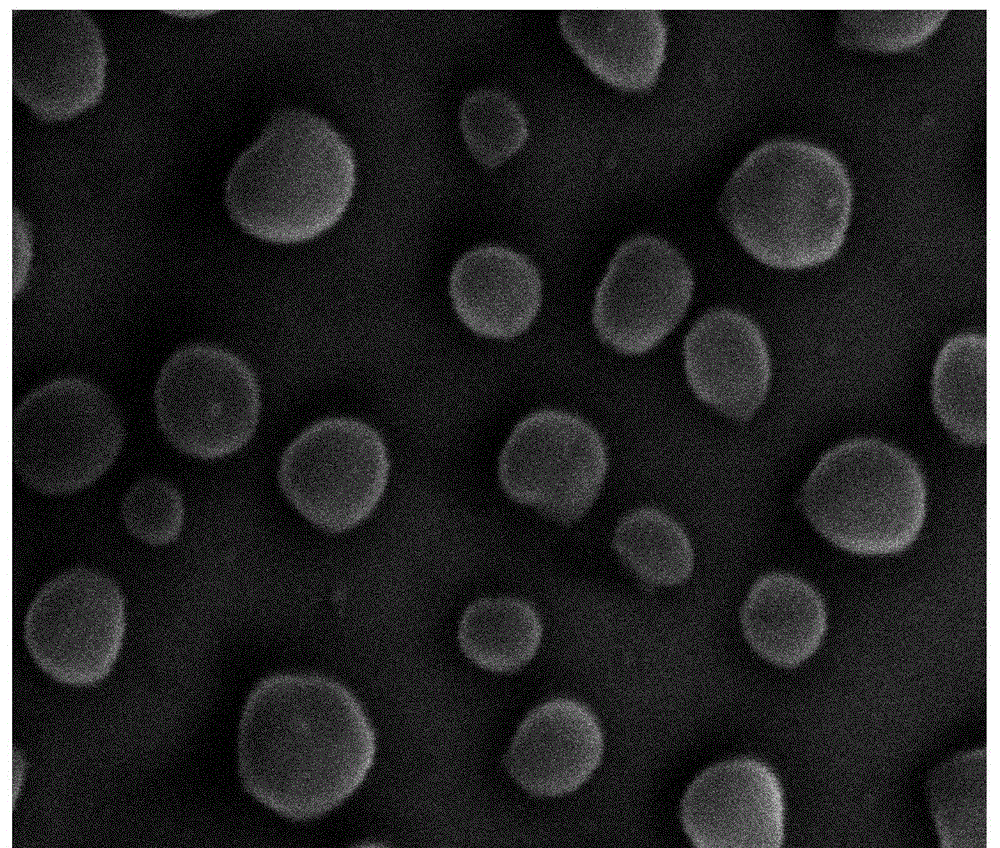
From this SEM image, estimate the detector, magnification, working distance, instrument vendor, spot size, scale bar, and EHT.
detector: ETD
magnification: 400 K X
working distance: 12 mm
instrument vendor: FEI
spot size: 3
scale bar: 300 nm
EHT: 20 kV